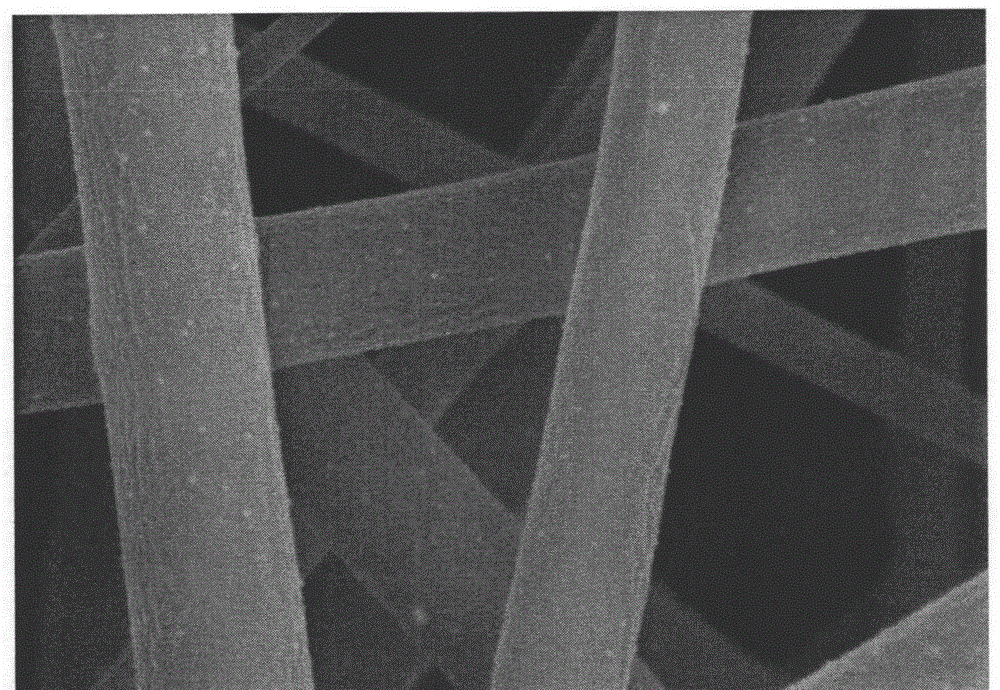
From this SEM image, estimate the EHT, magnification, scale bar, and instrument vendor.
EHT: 5 kV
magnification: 50 K X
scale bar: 1000 nm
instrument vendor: Hitachi